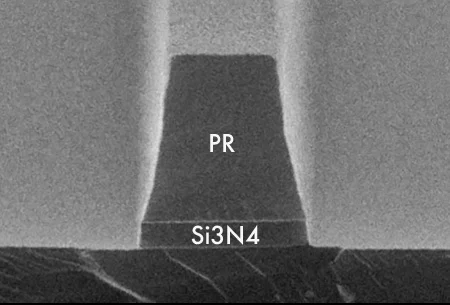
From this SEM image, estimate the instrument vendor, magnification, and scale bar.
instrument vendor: other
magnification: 25 K X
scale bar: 1000 nm